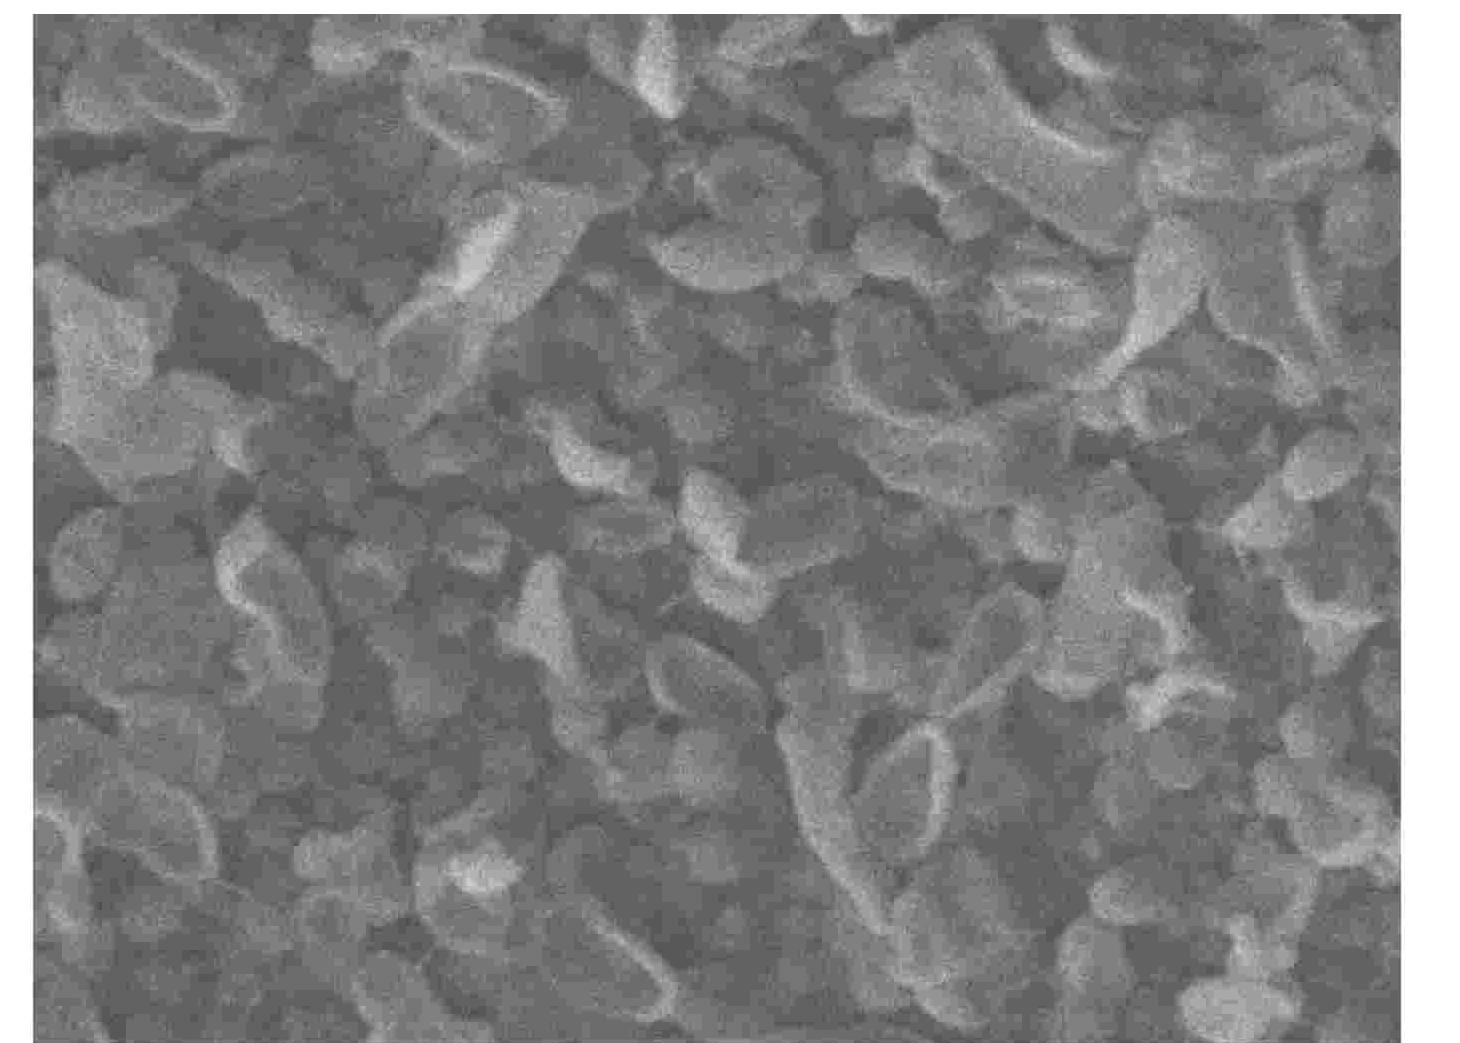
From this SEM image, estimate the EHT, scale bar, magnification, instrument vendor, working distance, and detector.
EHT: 5 kV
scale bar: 100 nm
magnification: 50 K X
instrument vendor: JEOL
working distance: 7.9 mm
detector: LEI